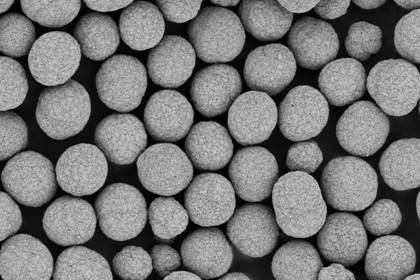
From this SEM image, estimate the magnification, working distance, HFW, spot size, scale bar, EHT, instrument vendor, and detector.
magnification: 1 K X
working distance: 4 mm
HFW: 127 µm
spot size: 8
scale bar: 40000 nm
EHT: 1.5 kV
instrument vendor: Thermo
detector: T1